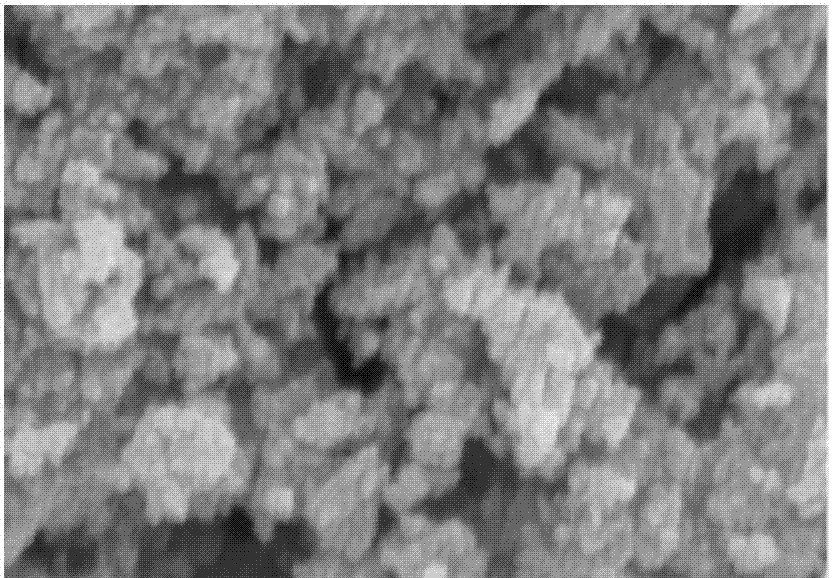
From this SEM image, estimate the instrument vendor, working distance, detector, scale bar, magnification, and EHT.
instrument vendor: Hitachi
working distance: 8.8 mm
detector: SE(M)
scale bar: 500 nm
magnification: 100 K X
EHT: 5 kV